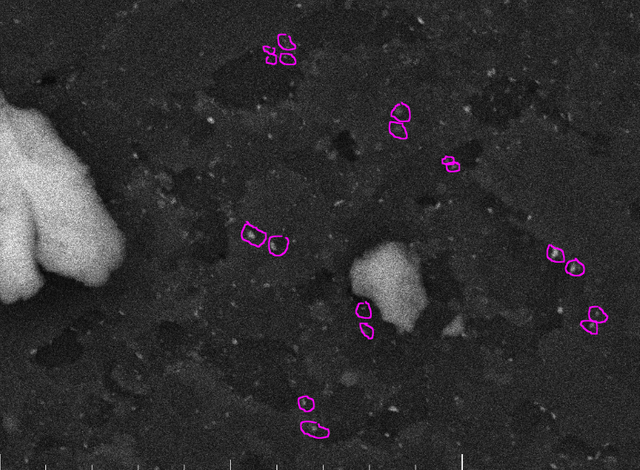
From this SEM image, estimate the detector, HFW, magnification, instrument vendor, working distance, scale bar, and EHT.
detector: SE, BSE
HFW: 13.8 µm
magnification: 20 K X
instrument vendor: Tescan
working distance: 15 mm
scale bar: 10000 nm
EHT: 15 kV